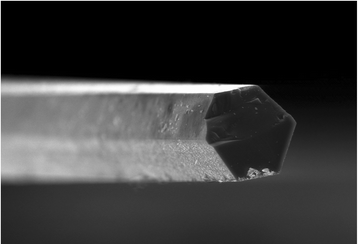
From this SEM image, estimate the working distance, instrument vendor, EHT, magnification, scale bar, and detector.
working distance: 7.9 mm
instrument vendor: Hitachi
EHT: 15 kV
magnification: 1 K X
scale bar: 50000 nm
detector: SE(U)